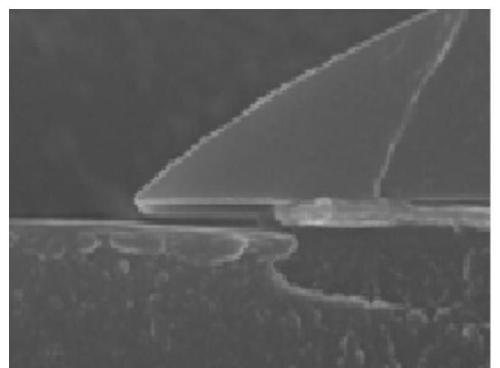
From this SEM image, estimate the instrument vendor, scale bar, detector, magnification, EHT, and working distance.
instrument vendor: Hitachi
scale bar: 1000 nm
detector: SE(U)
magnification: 50 K X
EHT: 15 kV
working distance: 11 mm